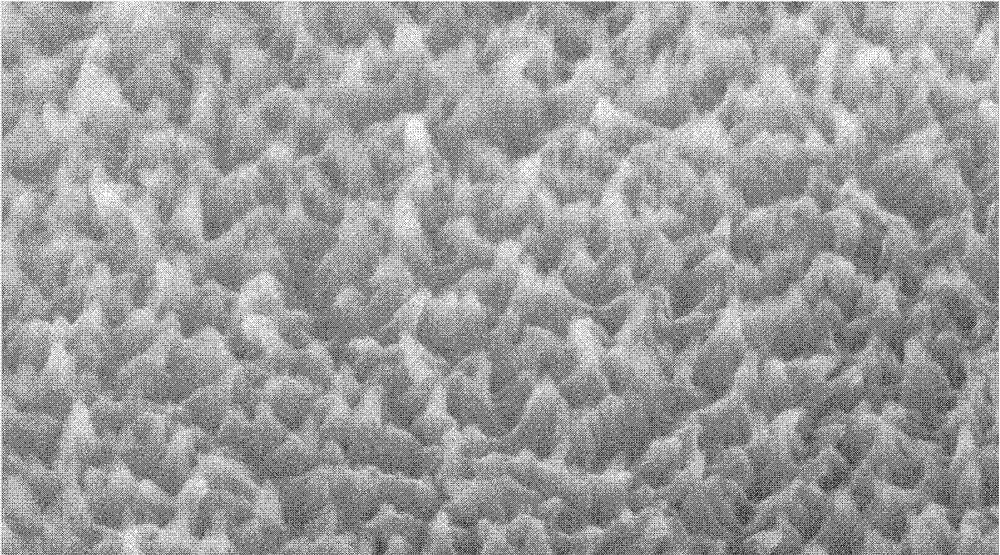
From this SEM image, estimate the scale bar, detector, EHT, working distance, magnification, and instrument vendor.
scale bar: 500 nm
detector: SE(M)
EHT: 6 kV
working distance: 6.8 mm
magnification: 80 K X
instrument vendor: Hitachi